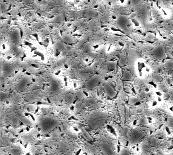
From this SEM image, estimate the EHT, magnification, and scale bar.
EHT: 20 kV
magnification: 1 K X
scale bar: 10000 nm